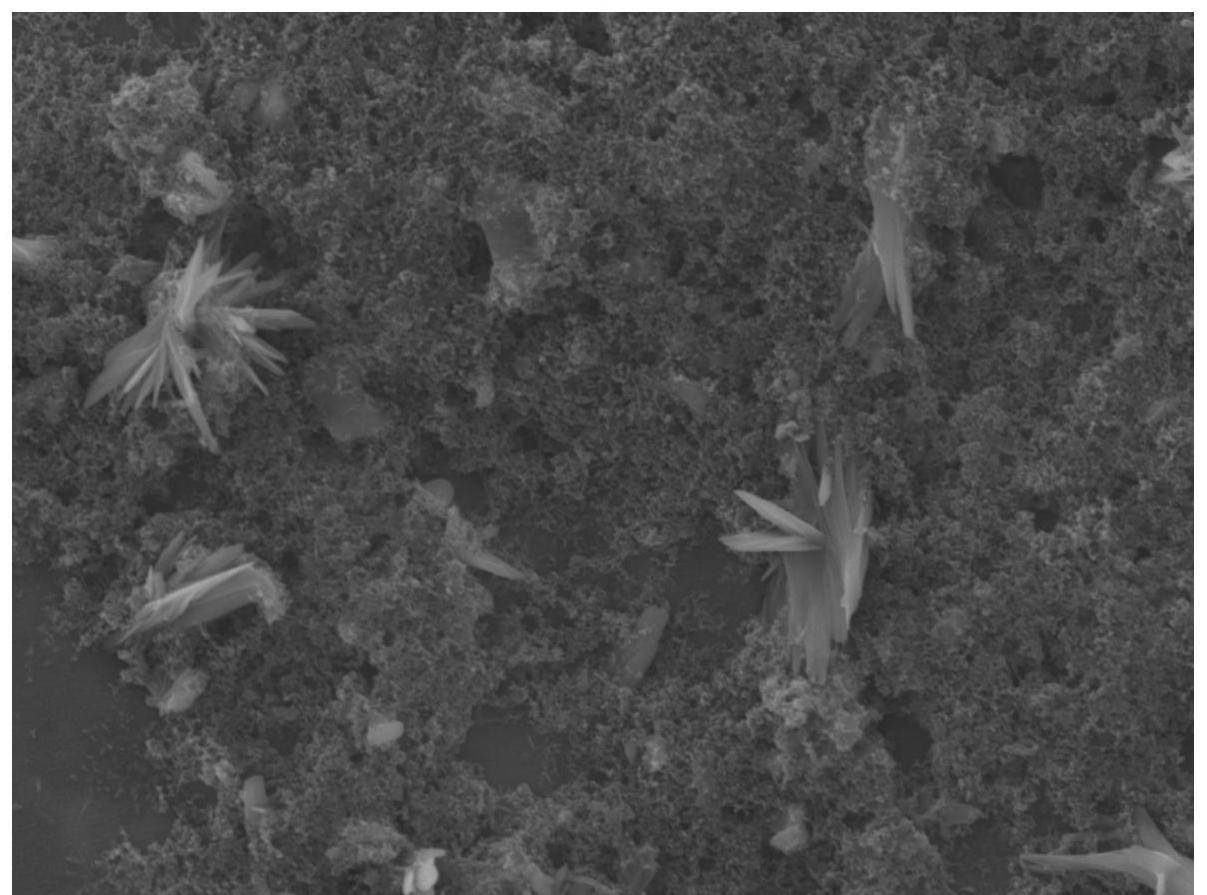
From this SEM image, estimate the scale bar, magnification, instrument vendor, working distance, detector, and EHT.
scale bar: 1000 nm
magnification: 5 K X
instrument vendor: JEOL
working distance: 9.7 mm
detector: LED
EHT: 10 kV